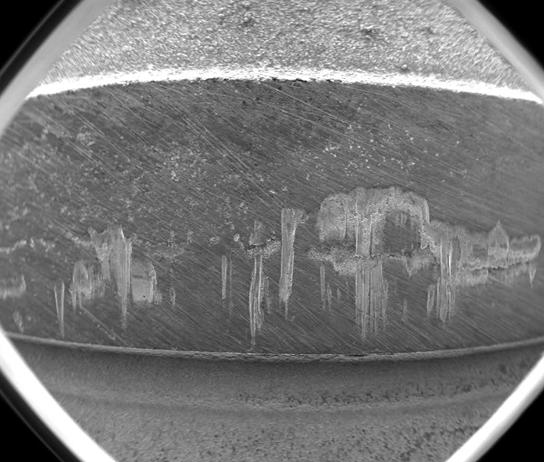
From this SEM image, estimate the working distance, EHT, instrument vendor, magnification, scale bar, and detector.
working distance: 10 mm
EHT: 20 kV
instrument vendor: FEI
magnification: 0.05 K X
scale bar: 2000000 nm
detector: ETD